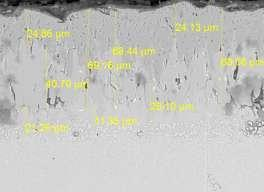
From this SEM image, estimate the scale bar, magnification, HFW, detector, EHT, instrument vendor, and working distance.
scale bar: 50000 nm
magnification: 1.5 K X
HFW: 184 µm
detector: BSE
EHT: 20 kV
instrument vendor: Tescan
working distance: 6.19 mm